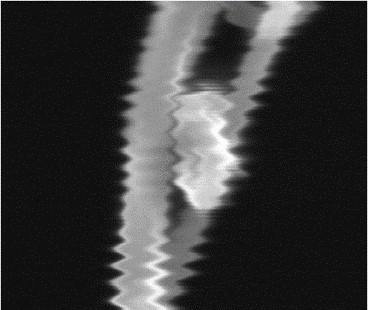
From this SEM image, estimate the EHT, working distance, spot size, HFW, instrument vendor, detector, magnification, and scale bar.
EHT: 30 kV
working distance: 10 mm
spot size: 7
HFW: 1.49 µm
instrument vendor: FEI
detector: ETD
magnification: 100 K X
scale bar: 500 nm